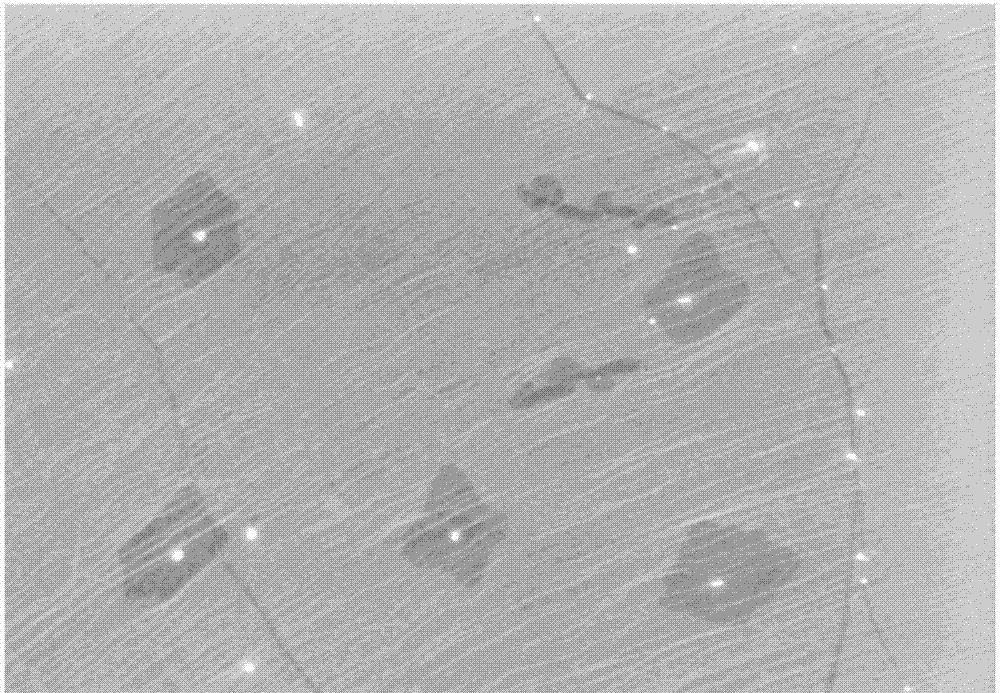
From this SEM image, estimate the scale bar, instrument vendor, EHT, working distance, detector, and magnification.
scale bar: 5000 nm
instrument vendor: Hitachi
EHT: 5 kV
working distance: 8.3 mm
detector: SE(M)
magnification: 11 K X